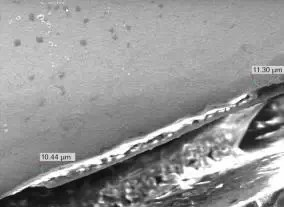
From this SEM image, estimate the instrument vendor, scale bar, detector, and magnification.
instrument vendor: JEOL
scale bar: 20000 nm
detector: SEI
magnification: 0.5 K X